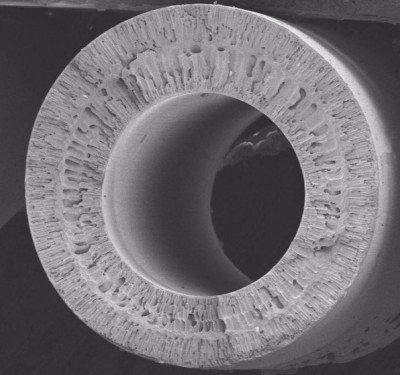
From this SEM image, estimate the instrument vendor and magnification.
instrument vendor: Hitachi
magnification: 0.07 K X